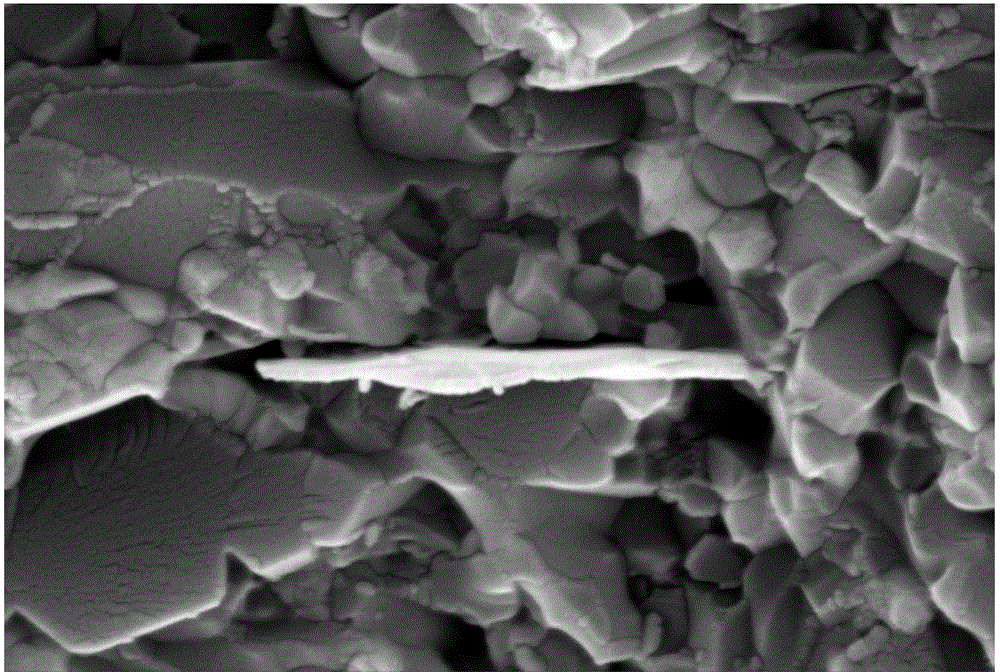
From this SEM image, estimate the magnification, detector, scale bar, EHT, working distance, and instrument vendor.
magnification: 20 K X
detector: SE2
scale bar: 200 nm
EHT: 15 kV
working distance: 9.2 mm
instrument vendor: Zeiss